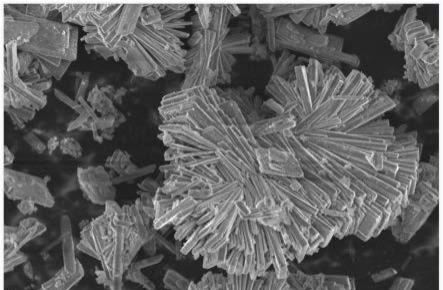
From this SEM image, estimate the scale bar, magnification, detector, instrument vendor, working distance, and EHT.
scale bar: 5000 nm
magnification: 2 K X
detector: InLens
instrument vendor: Zeiss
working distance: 5 mm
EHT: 5 kV